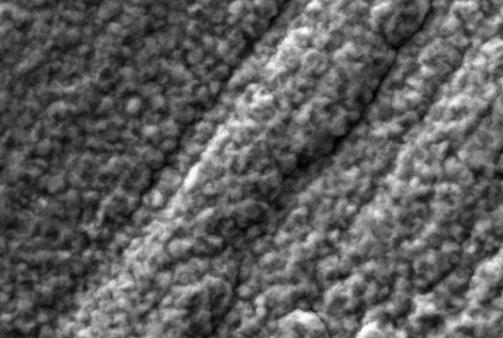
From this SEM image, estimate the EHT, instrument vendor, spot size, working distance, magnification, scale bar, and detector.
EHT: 20 kV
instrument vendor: JEOL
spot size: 40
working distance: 9 mm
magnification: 3 K X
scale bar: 5000 nm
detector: SEI